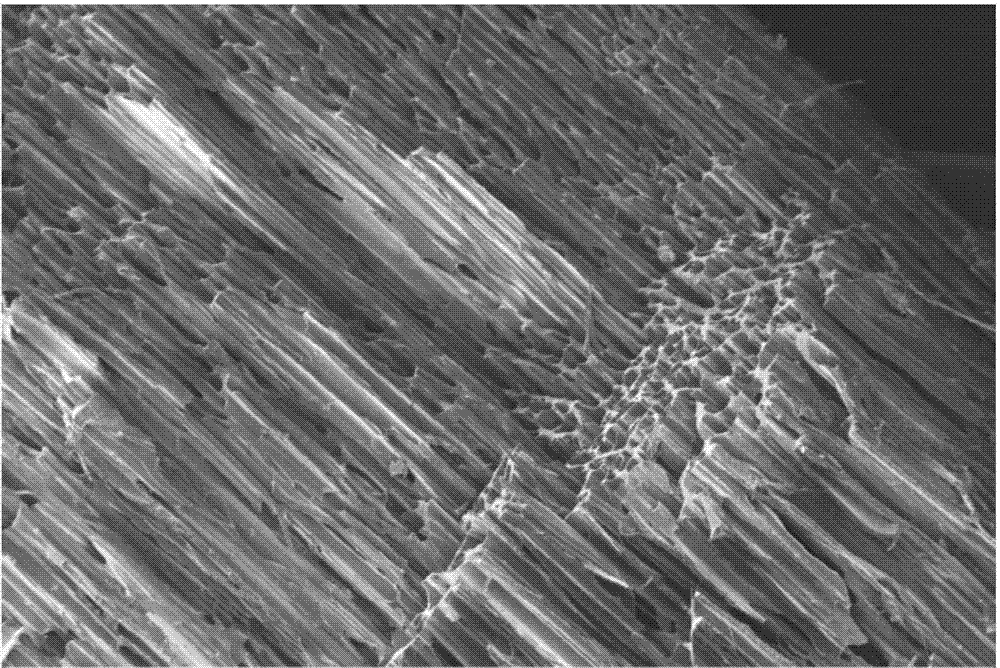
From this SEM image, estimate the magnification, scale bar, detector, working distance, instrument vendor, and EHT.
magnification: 0.025 K X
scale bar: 200000 nm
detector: SE2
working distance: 18.4 mm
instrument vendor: Zeiss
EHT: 10 kV